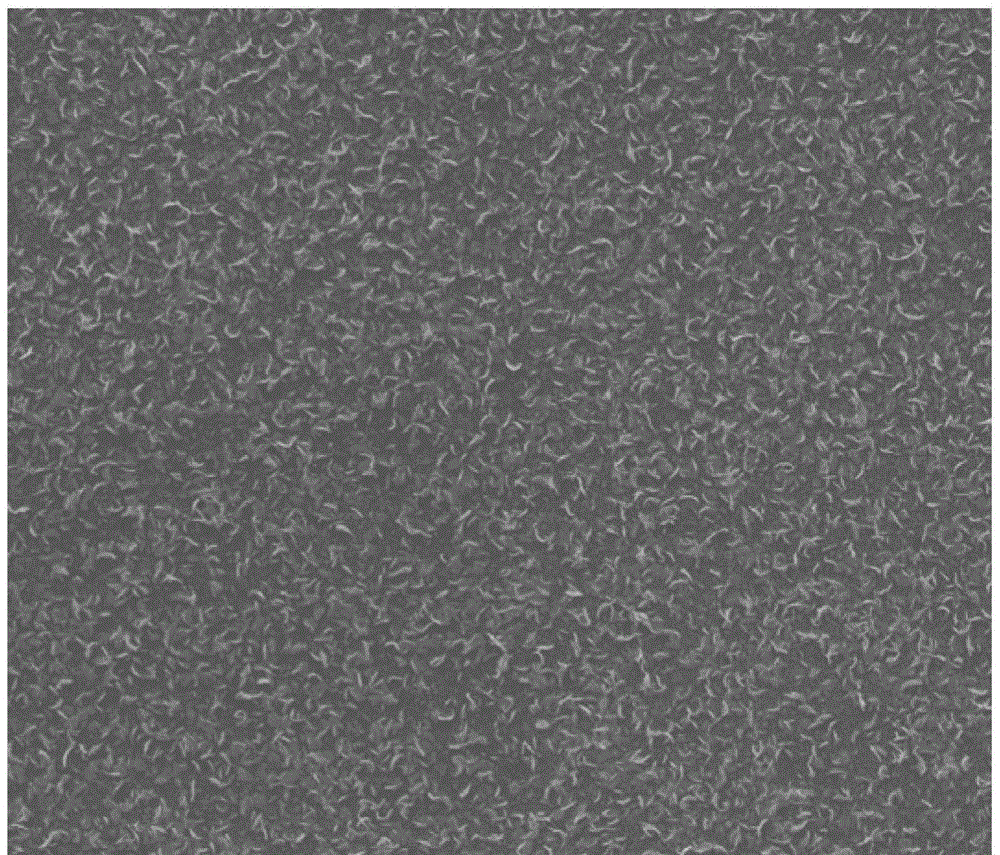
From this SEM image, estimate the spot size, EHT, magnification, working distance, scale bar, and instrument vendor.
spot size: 3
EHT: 20 kV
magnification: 50 K X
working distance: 9.7 mm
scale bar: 2000 nm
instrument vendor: FEI